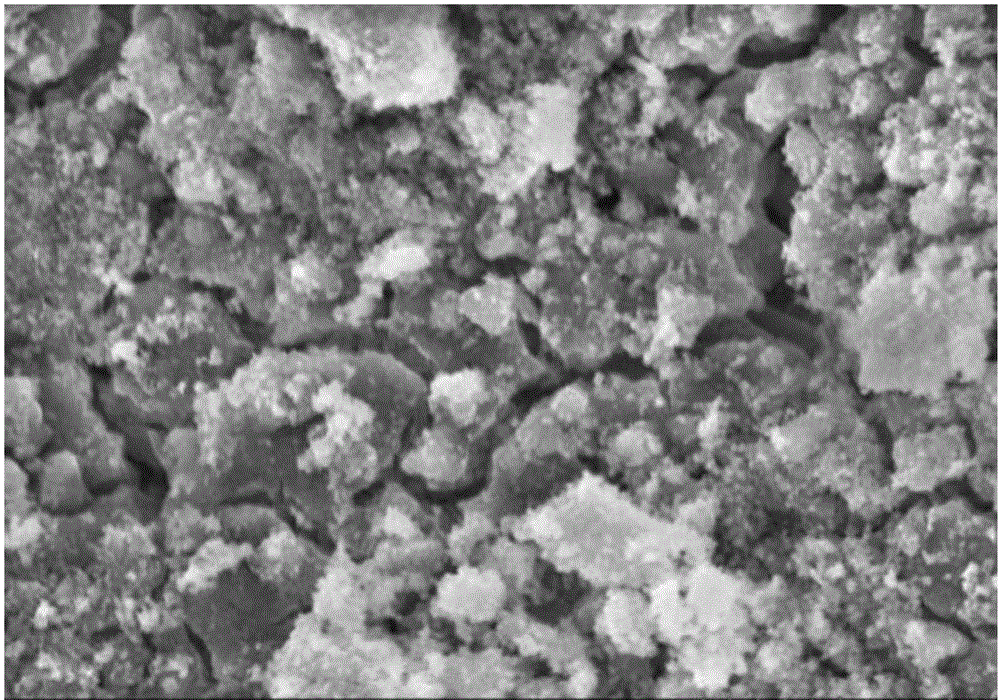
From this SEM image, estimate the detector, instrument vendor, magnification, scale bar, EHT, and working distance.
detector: SE(M)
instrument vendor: Hitachi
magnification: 7 K X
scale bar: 5000 nm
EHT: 10 kV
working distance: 10.2 mm